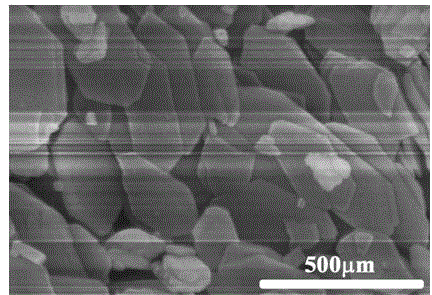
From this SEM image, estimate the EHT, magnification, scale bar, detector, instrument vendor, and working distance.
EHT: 5 kV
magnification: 100 K X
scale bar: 500 nm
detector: SE(UL)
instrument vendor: Hitachi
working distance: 11.6 mm